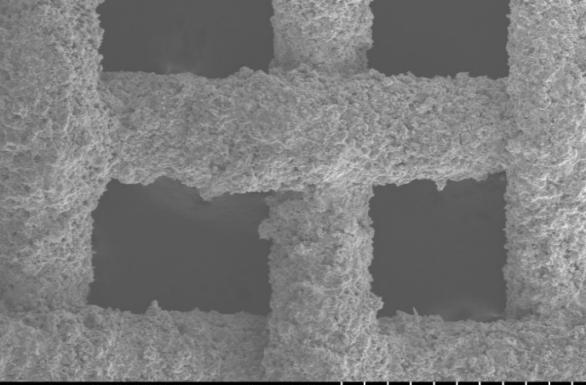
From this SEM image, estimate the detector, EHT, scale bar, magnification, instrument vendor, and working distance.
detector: LM(L)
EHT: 20 kV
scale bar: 500000 nm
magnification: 0.1 K X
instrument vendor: Hitachi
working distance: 10.2 mm